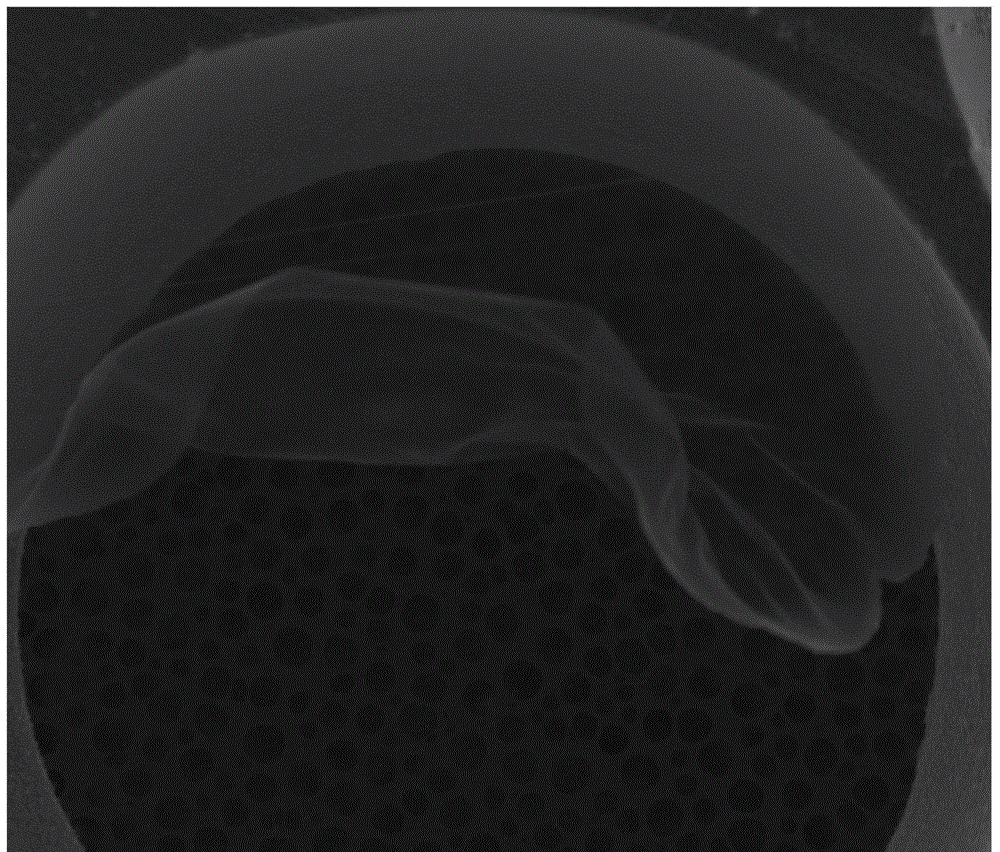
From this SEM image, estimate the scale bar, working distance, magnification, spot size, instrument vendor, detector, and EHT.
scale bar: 30000 nm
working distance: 10.7 mm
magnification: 1.5 K X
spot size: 3.5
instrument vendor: FEI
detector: SE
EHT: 20 kV